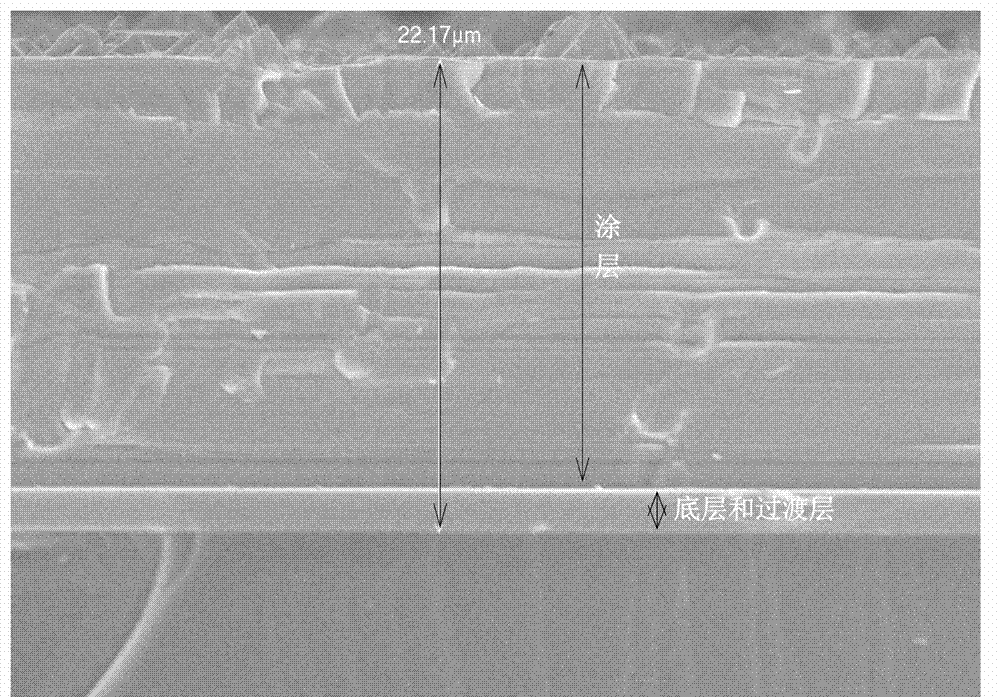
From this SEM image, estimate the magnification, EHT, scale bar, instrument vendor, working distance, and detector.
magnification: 2 K X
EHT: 10 kV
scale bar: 10000 nm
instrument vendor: JEOL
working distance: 7.9 mm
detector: SEI